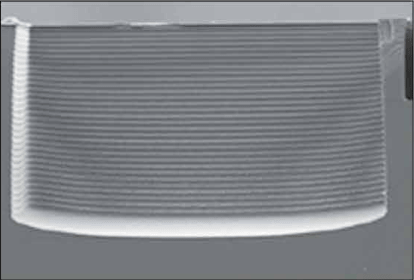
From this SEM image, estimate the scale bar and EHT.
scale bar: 50000 nm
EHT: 7.5 kV